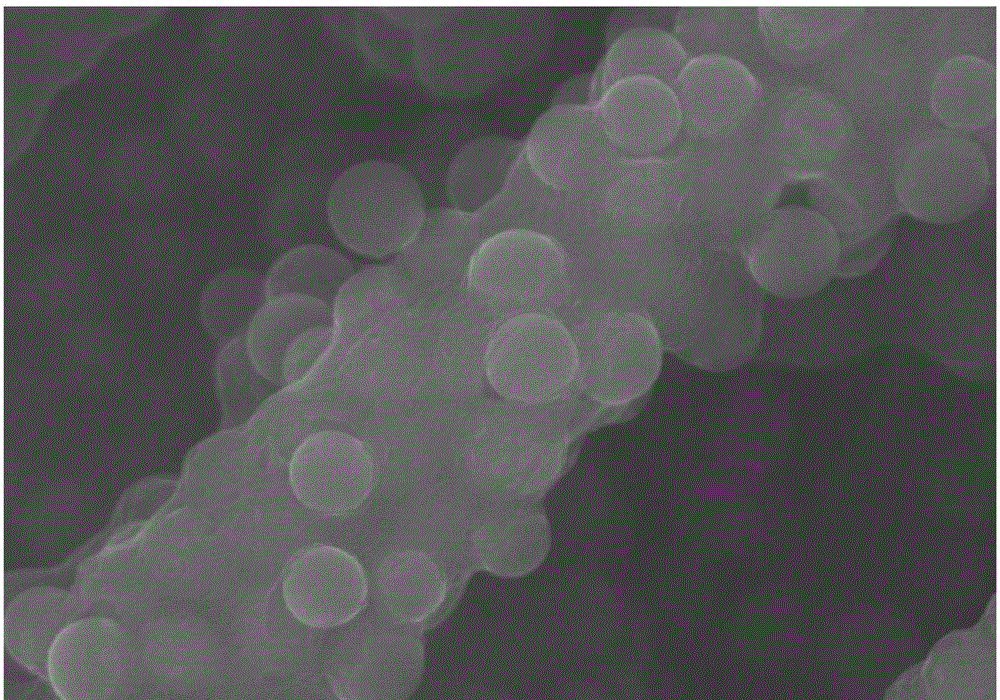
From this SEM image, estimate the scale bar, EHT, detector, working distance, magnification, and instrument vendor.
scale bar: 1000 nm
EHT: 15 kV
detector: SE(U)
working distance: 8.4 mm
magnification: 40 K X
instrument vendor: Hitachi